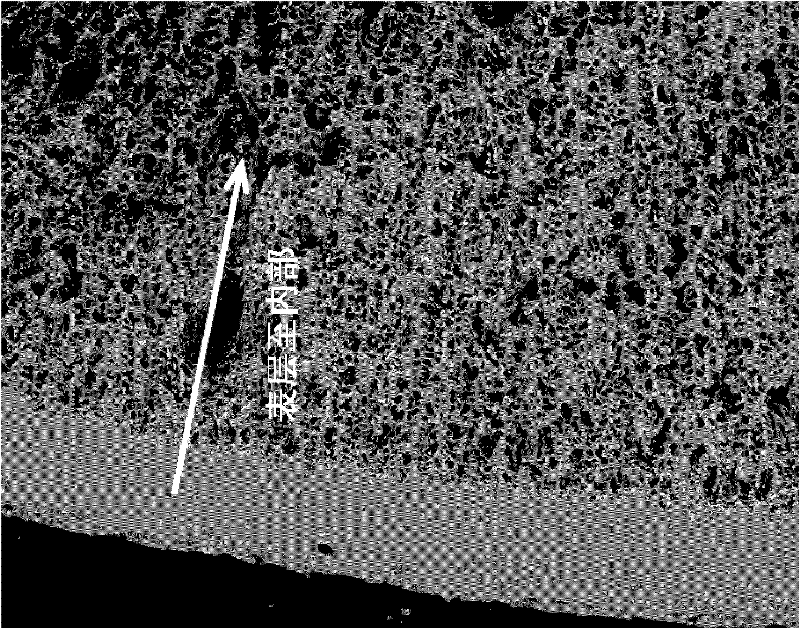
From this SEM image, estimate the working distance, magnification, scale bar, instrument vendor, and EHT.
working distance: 9.7 mm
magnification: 0.09 K X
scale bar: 1000000 nm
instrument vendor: FEI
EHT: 20 kV